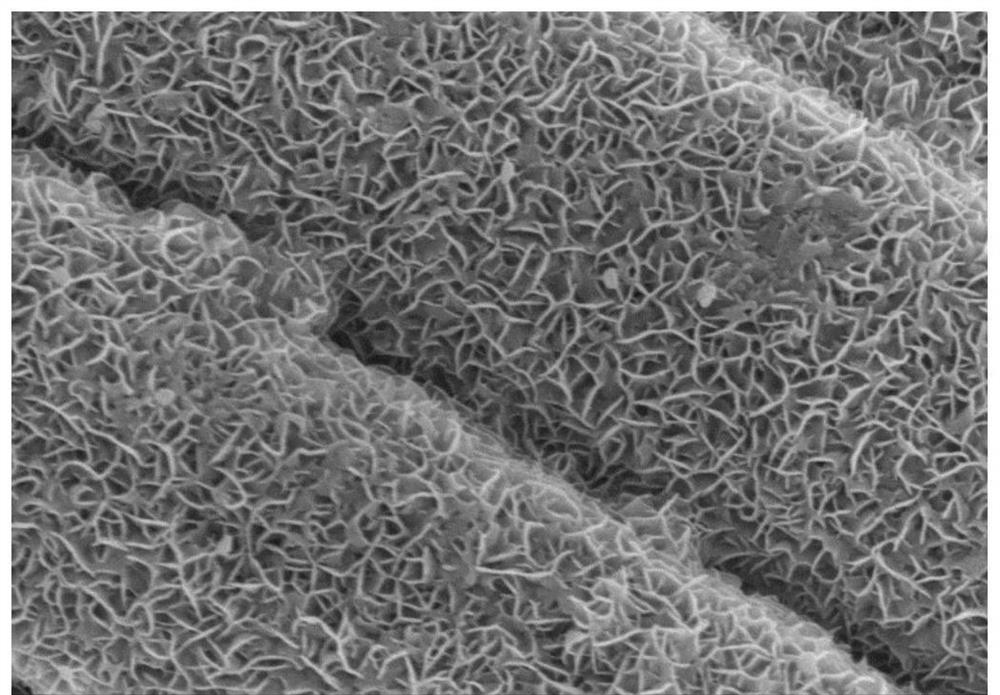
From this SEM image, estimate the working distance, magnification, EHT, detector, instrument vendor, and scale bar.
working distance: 9.9 mm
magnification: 30 K X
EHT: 5 kV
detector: SE(M)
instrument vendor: Hitachi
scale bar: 1000 nm